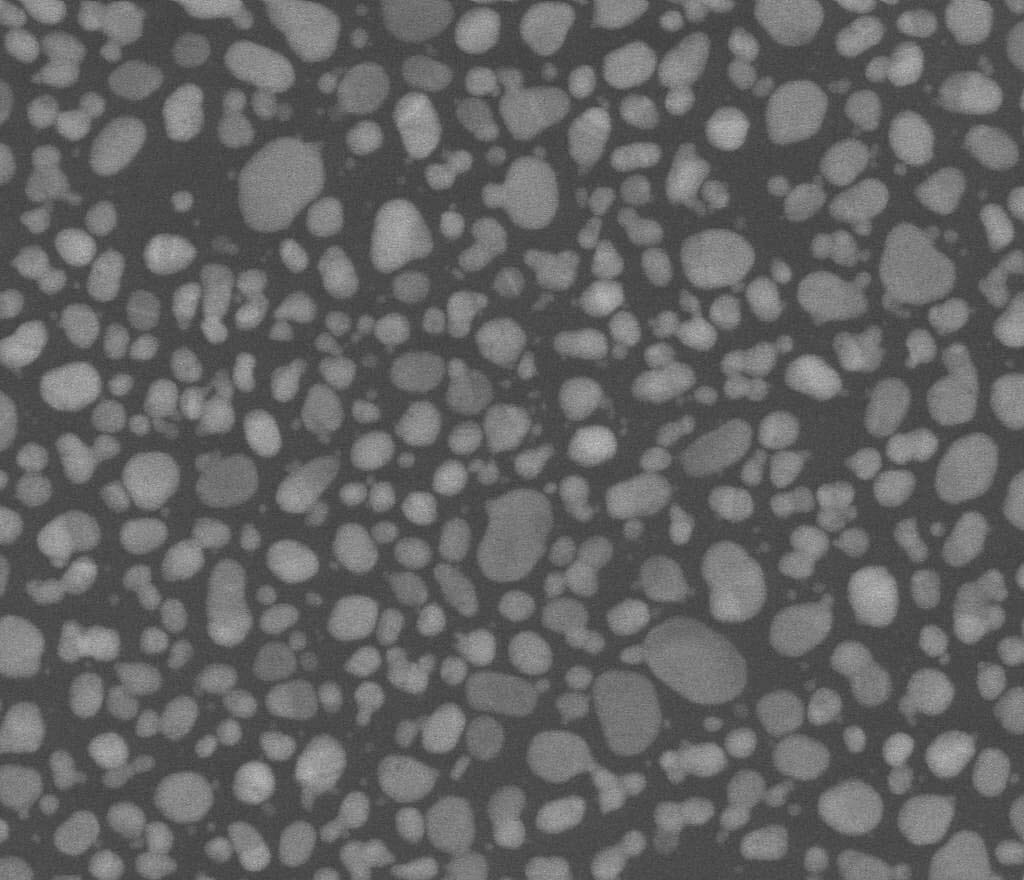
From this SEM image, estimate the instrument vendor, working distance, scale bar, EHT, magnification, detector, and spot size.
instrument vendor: FEI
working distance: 6.4 mm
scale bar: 300 nm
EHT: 30 kV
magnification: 200 K X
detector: BSED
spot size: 1